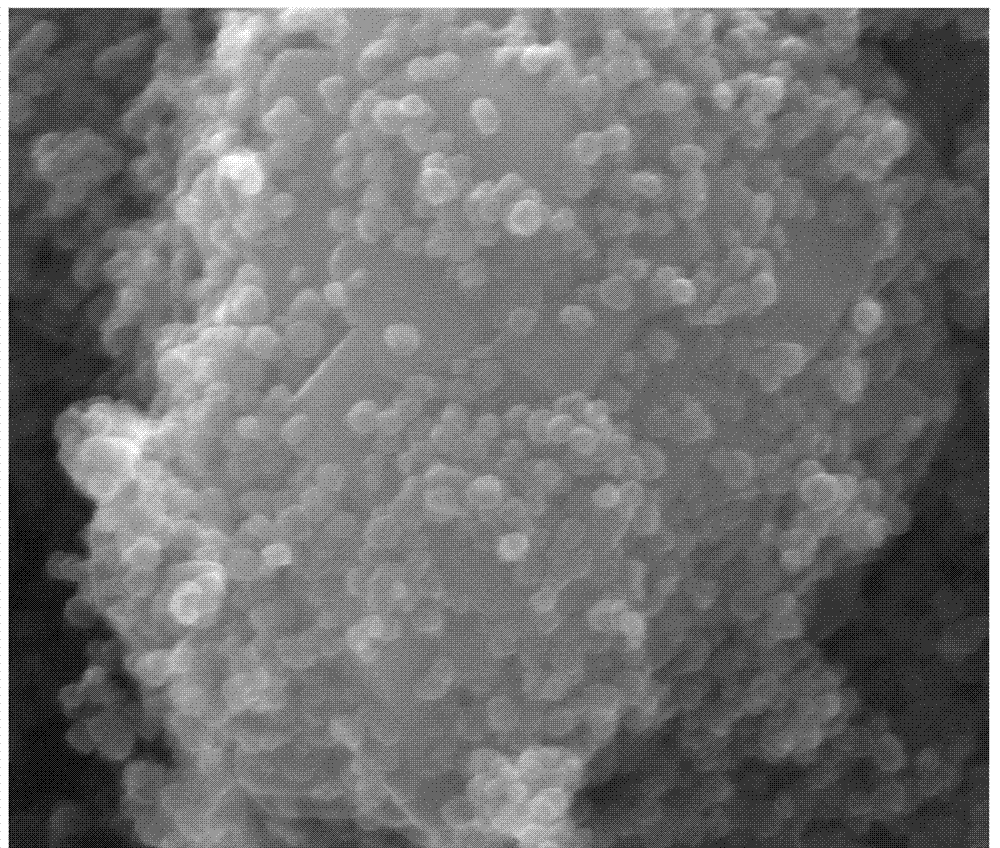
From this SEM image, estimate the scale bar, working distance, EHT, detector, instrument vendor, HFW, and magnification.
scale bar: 500 nm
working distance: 9.8 mm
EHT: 30 kV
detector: ETD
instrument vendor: FEI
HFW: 1.59 µm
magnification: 80 K X